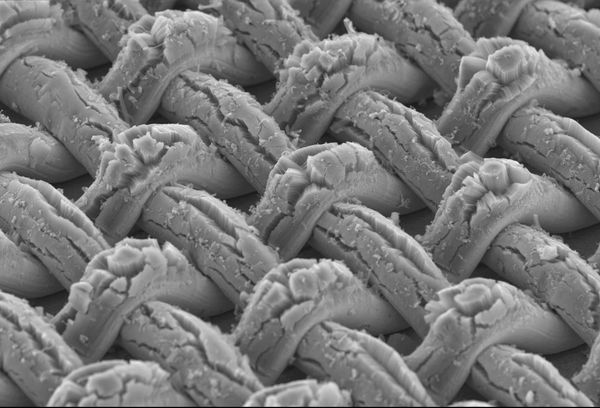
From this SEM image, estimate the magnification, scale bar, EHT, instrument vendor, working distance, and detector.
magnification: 0.1 K X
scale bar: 500000 nm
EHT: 10 kV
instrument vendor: Hitachi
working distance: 11.7 mm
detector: SE